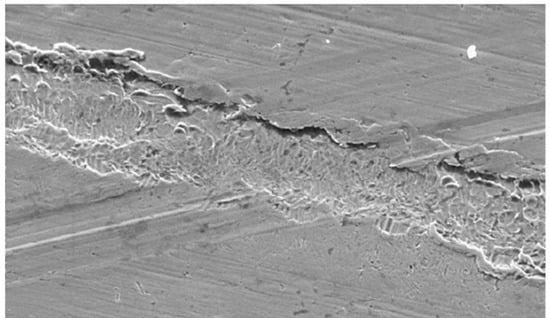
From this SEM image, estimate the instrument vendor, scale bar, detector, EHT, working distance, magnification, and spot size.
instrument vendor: FEI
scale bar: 10000 nm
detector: SE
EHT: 10 kV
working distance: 14.1 mm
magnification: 5 K X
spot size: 3